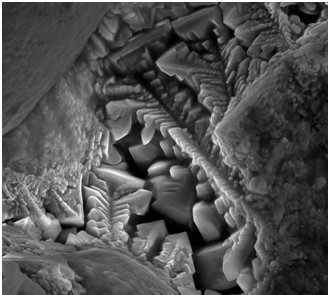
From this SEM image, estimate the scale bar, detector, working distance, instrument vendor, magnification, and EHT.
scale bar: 3000 nm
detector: SE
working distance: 9.2 mm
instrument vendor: other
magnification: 10 K X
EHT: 10 kV